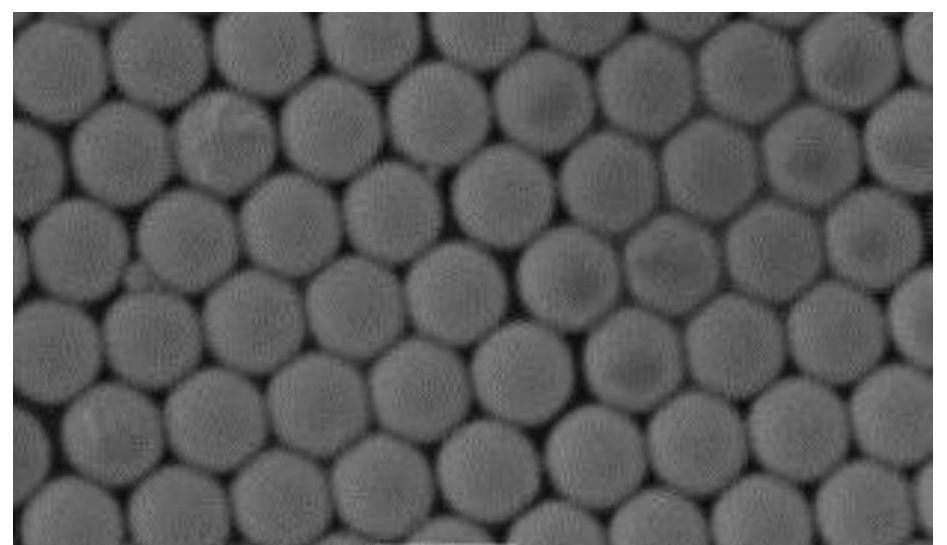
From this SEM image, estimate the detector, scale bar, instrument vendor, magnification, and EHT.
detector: SEI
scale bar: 1000 nm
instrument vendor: JEOL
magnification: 20 K X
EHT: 15 kV